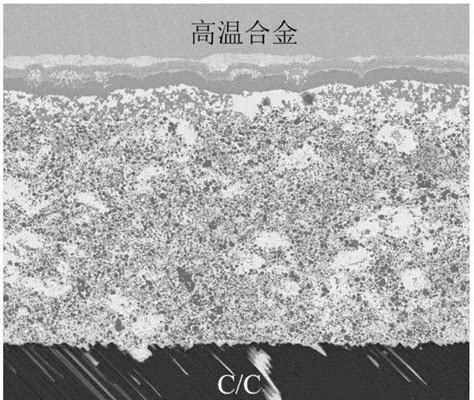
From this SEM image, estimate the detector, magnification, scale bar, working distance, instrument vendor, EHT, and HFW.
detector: BSED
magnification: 0.4 K X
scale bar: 200000 nm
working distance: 12.2 mm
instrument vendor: FEI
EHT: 20 kV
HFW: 746 µm